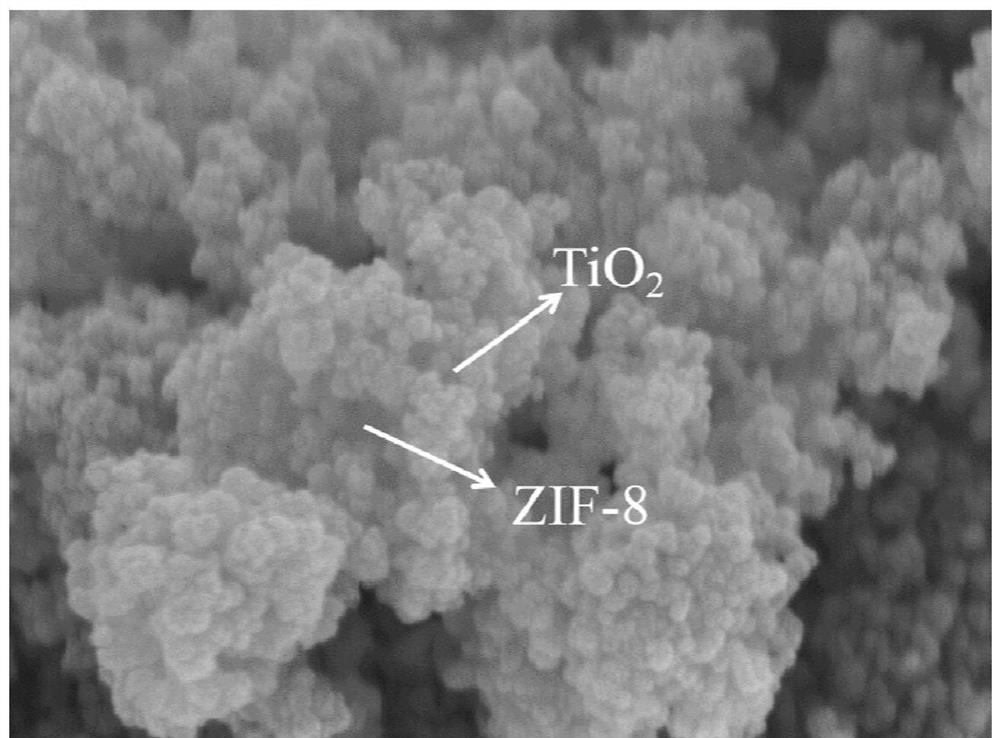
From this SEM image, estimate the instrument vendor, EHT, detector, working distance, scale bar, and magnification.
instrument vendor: Hitachi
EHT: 5 kV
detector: SE(U)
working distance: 8.3 mm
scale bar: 500 nm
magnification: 100 K X